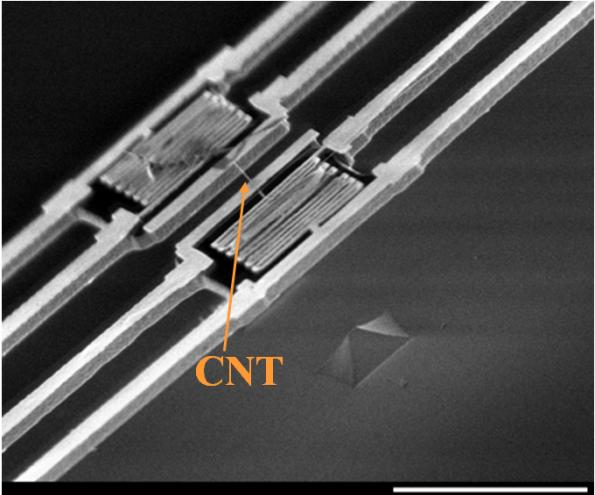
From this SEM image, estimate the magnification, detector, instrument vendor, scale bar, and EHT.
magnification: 2.5 K X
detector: SEI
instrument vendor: JEOL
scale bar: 10000 nm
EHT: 1 kV